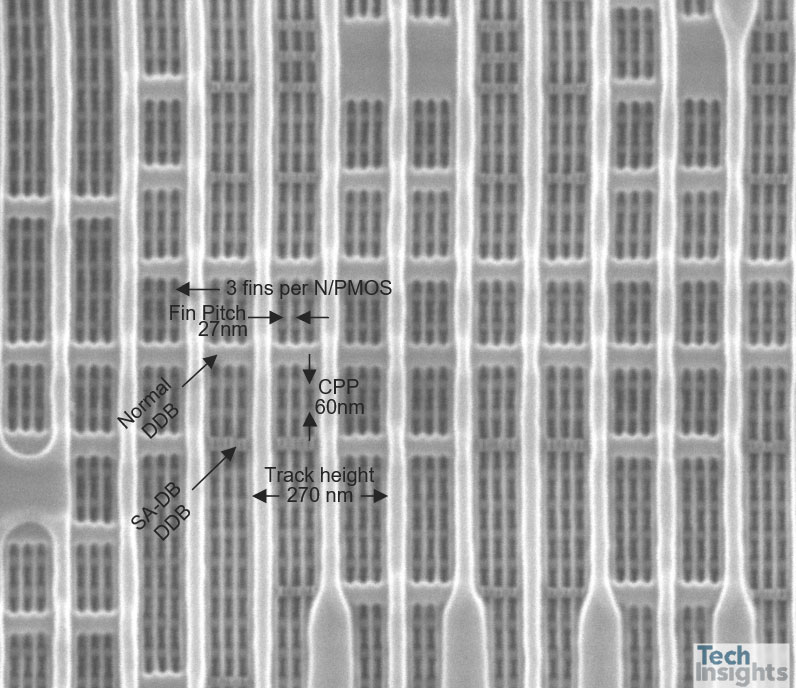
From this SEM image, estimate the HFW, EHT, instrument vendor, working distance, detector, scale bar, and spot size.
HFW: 1.59 µm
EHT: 10 kV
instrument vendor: FEI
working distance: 10.6 mm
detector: ETD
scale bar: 500 nm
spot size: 2.5